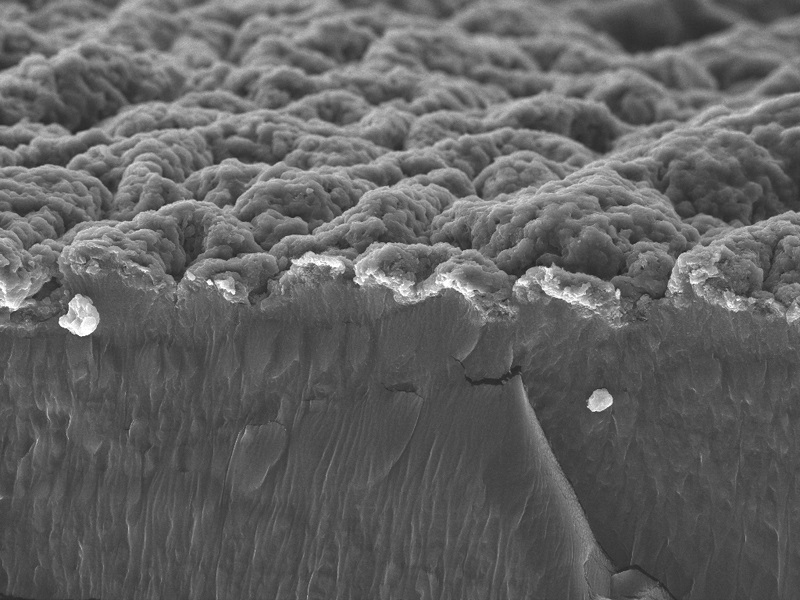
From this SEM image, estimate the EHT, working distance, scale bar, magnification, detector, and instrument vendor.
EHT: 20 kV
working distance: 13.21 mm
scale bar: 50000 nm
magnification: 1 K X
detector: SE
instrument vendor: Tescan